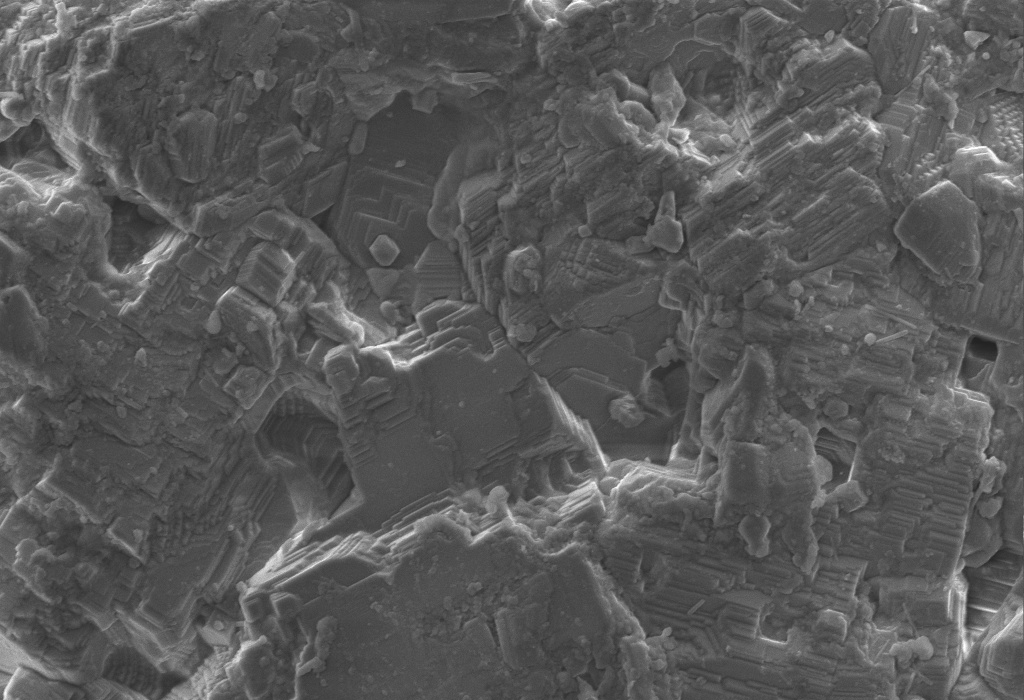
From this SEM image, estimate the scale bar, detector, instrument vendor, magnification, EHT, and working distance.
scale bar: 10000 nm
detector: SE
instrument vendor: other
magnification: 2 K X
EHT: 10 kV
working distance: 10.3 mm